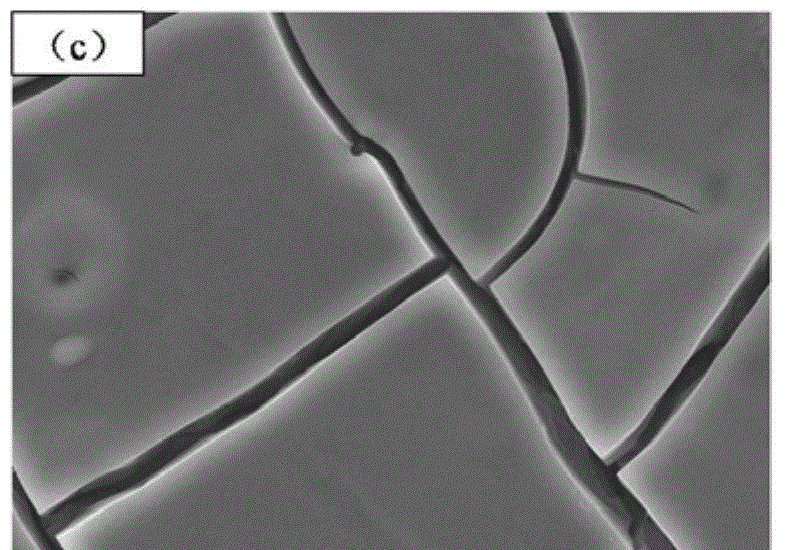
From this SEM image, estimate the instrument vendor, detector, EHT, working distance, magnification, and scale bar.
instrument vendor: JEOL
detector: SEI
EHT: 15 kV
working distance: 7.2 mm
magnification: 3 K X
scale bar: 1000 nm